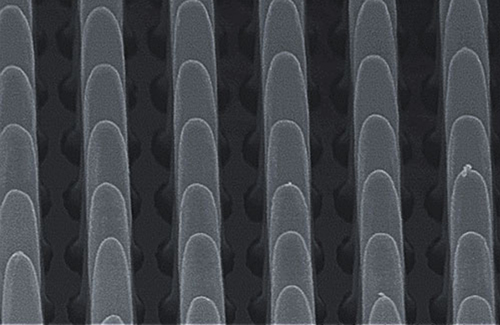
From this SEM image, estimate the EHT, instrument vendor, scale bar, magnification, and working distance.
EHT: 15 kV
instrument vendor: Hitachi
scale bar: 2000 nm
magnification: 20 K X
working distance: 13.2 mm